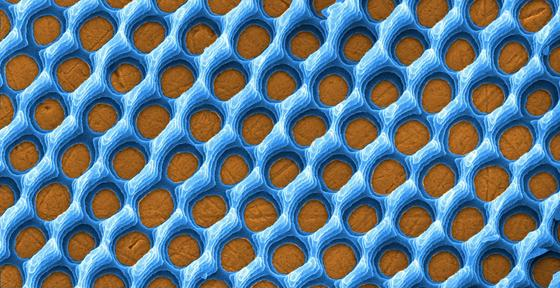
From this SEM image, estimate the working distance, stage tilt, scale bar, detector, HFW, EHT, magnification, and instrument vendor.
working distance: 4 mm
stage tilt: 20°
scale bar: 4000 nm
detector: TLD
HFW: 8.53 µm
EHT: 5 kV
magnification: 15 K X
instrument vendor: FEI